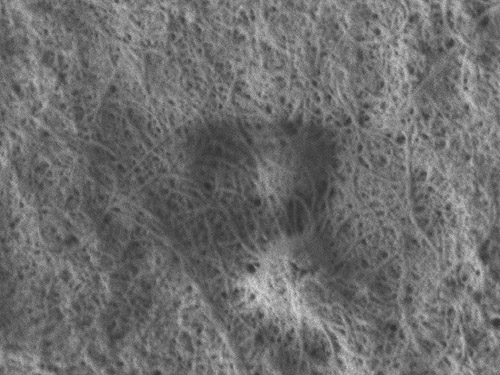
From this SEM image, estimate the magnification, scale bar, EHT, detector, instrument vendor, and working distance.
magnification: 50 K X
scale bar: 100 nm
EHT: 1 kV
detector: LEI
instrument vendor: JEOL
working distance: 7.8 mm